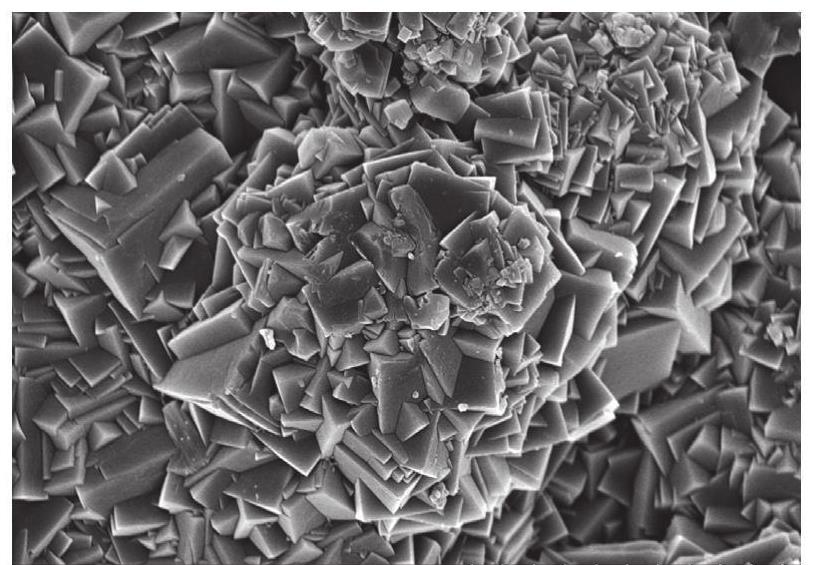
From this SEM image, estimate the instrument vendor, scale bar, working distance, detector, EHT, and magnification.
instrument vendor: Hitachi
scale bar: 5000 nm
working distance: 9.1 mm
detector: SE(U)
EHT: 5 kV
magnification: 10 K X